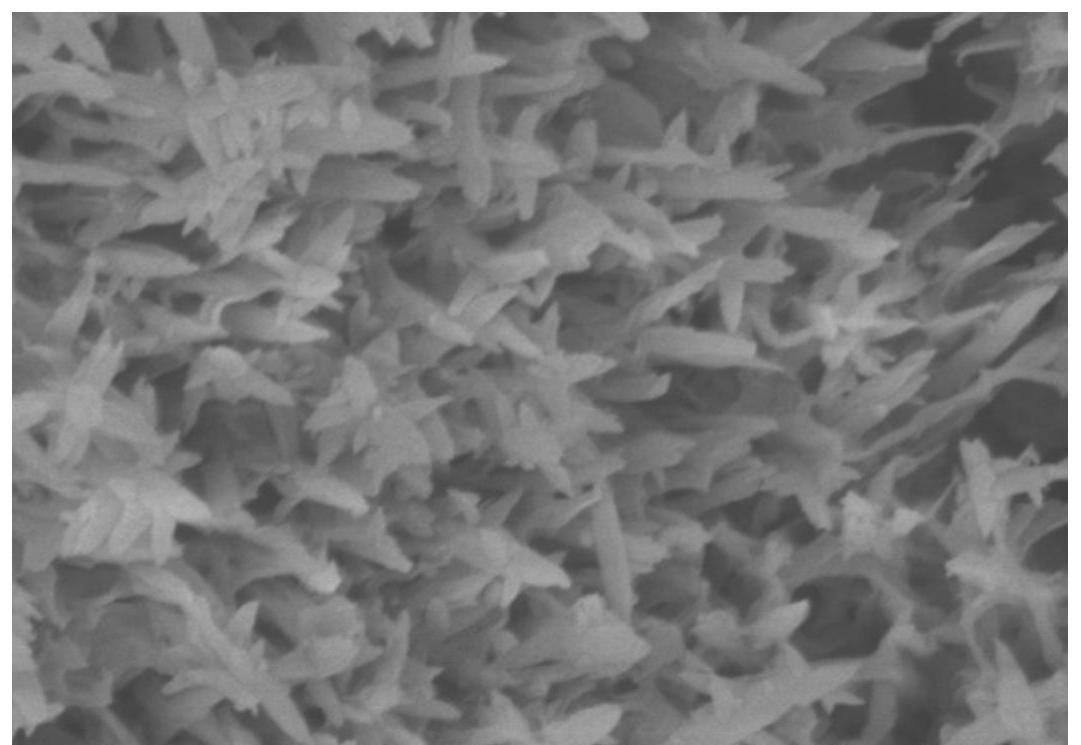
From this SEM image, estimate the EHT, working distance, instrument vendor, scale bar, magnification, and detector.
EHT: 5 kV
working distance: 8.1 mm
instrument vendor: Hitachi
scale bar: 1000 nm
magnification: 50 K X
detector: SE(M)